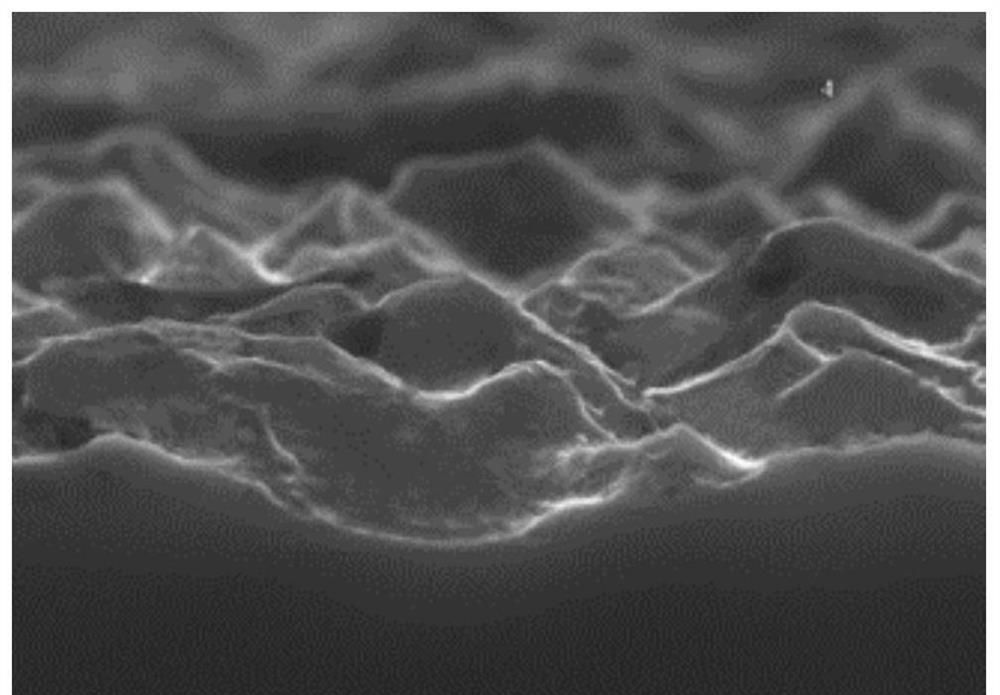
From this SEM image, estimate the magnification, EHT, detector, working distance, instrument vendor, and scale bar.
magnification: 11 K X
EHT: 15 kV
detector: SE(U)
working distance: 8.7 mm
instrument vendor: Hitachi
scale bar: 5000 nm